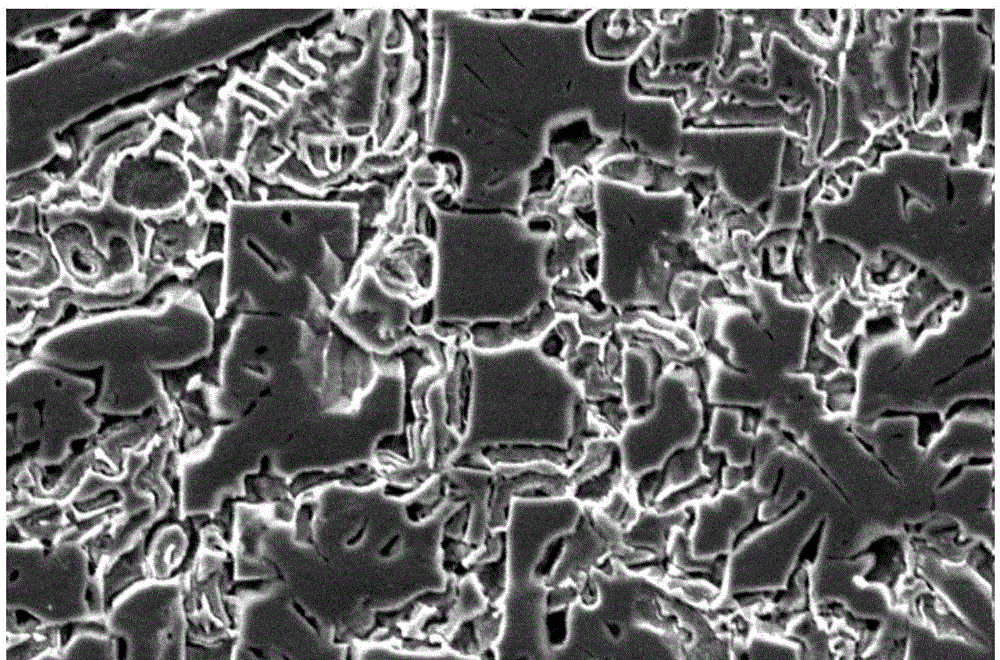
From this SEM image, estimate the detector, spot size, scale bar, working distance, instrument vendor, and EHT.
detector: SE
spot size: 5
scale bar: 20000 nm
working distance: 10.4 mm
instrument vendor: FEI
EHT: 20 kV